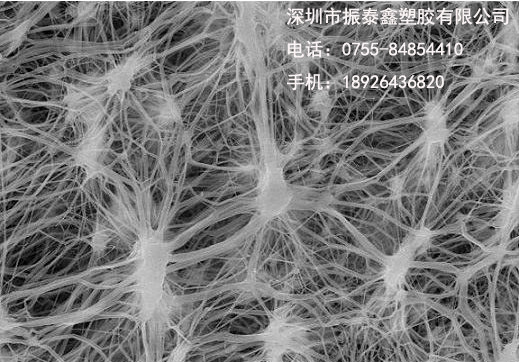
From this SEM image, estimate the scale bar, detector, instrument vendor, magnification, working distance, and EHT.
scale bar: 10000 nm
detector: SE(M)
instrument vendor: Hitachi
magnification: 5 K X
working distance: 8.2 mm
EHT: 15 kV